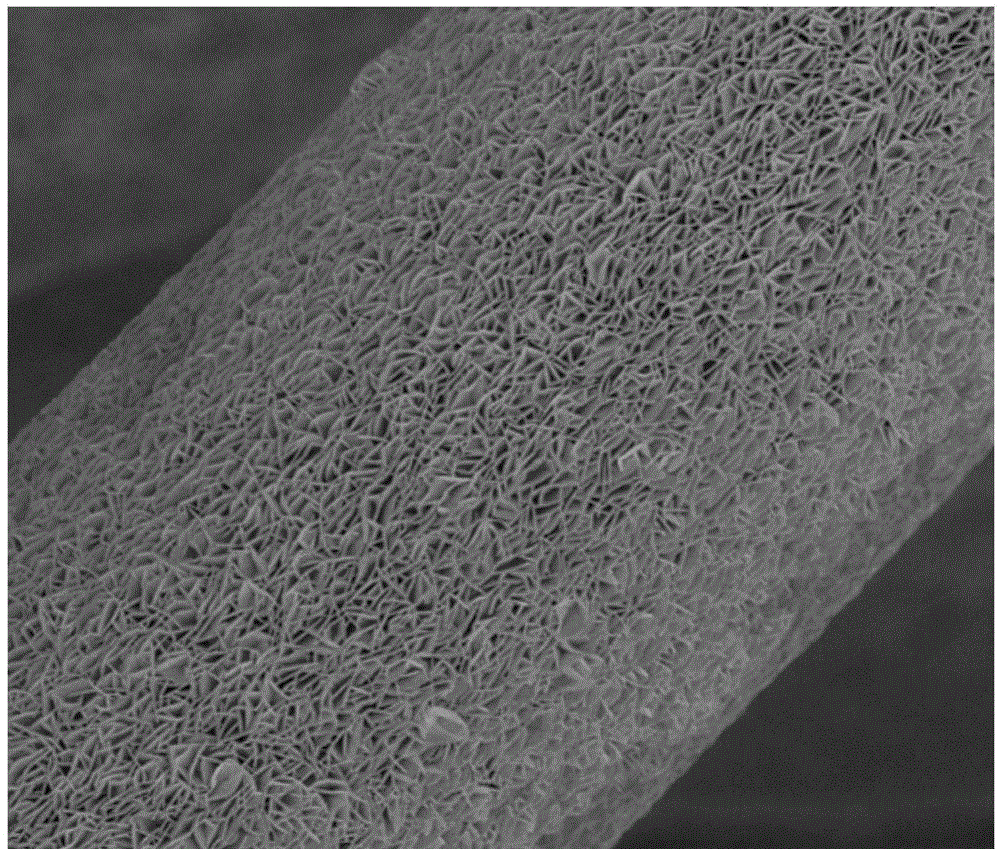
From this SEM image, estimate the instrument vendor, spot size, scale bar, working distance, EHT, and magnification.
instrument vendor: FEI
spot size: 3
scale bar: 4000 nm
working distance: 5 mm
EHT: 3 kV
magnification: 20 K X